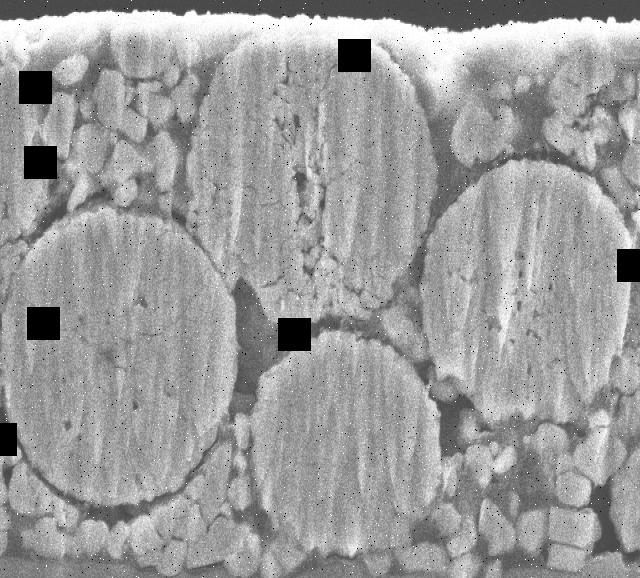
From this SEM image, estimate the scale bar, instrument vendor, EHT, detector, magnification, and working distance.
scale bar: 5000 nm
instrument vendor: JEOL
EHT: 15 kV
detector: SEI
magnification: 3.5 K X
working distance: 8 mm